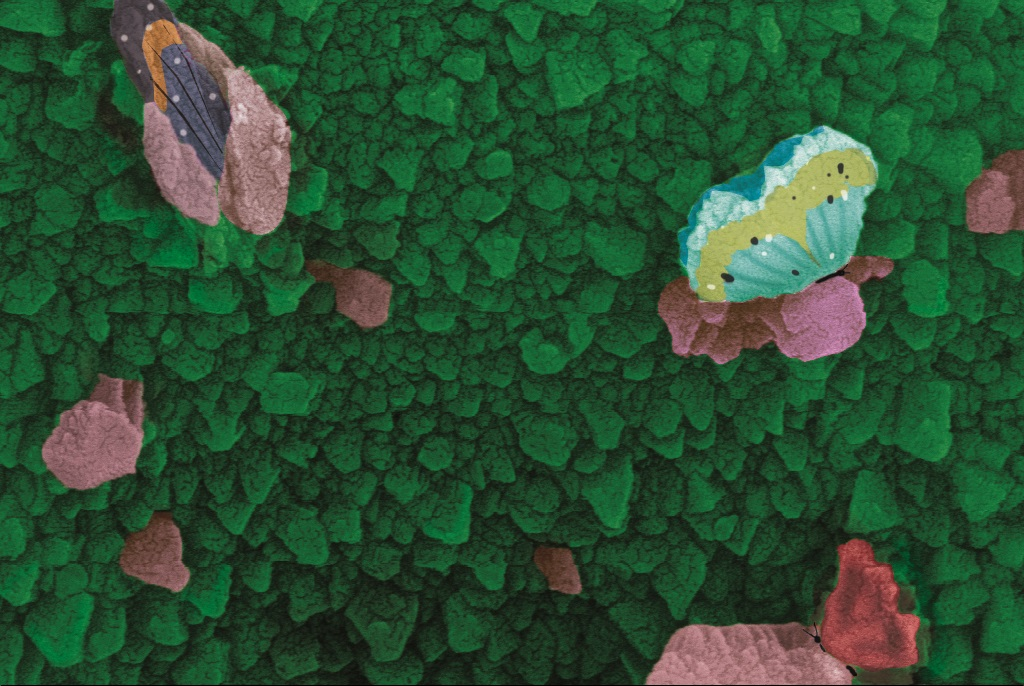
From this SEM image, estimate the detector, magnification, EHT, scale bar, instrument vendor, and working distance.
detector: RBSD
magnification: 100 K X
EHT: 20 kV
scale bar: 200 nm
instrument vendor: Zeiss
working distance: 11 mm